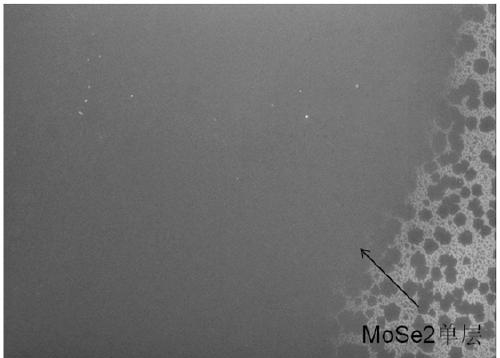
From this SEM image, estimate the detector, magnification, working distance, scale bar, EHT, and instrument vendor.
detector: ETD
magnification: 4.955 K X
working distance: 3.5 mm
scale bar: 30000 nm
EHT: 10 kV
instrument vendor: FEI